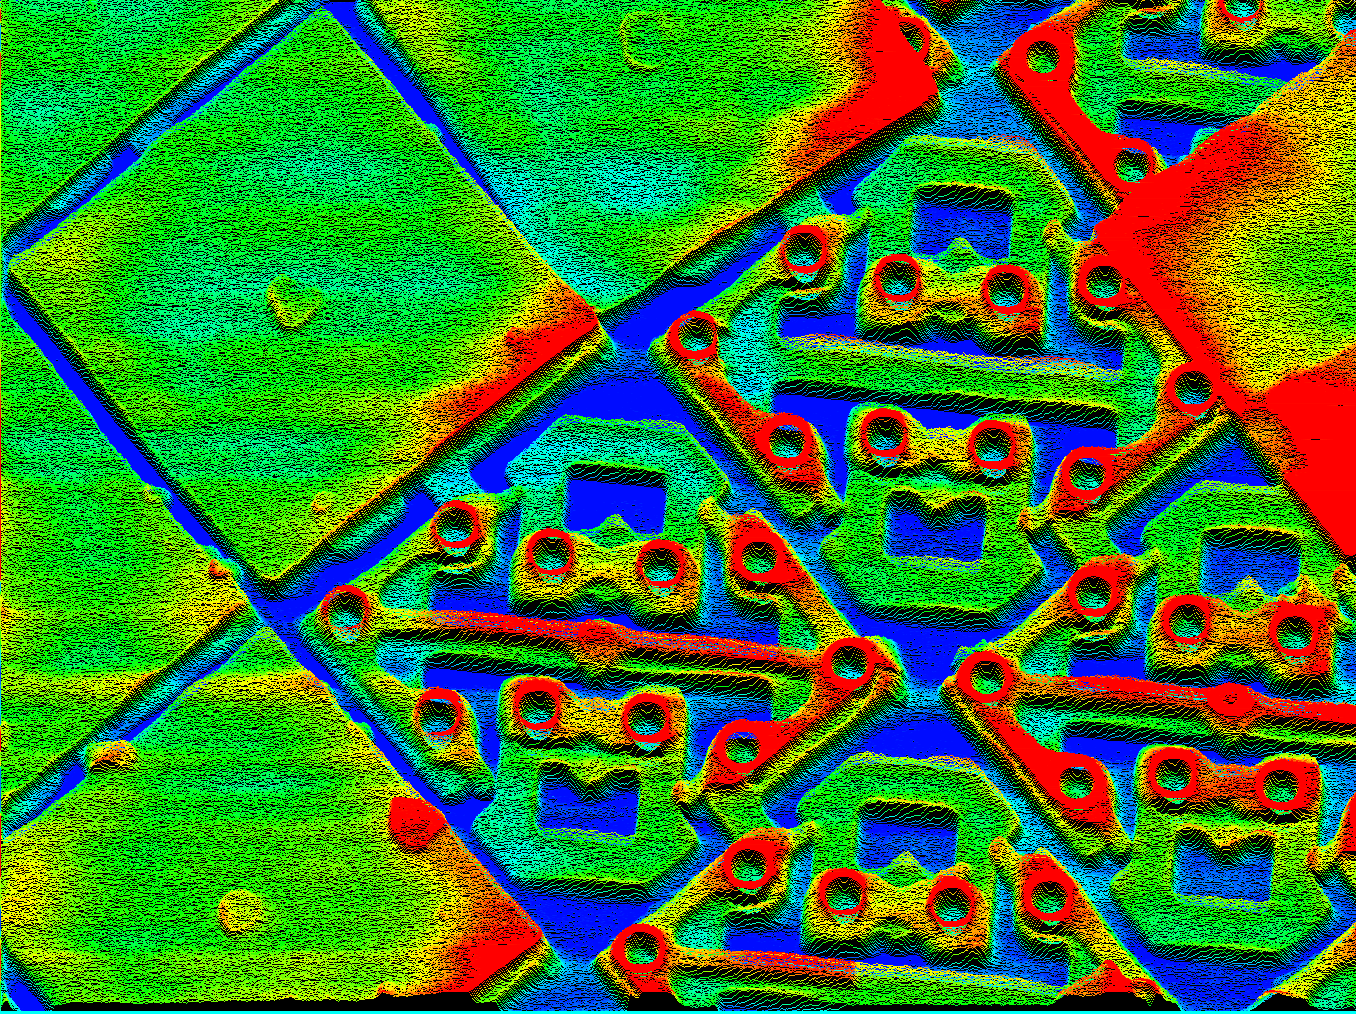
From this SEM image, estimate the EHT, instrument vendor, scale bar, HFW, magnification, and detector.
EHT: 15 kV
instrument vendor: other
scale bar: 3330 nm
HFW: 40 µm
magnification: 3 K X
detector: SE +Y-МОД